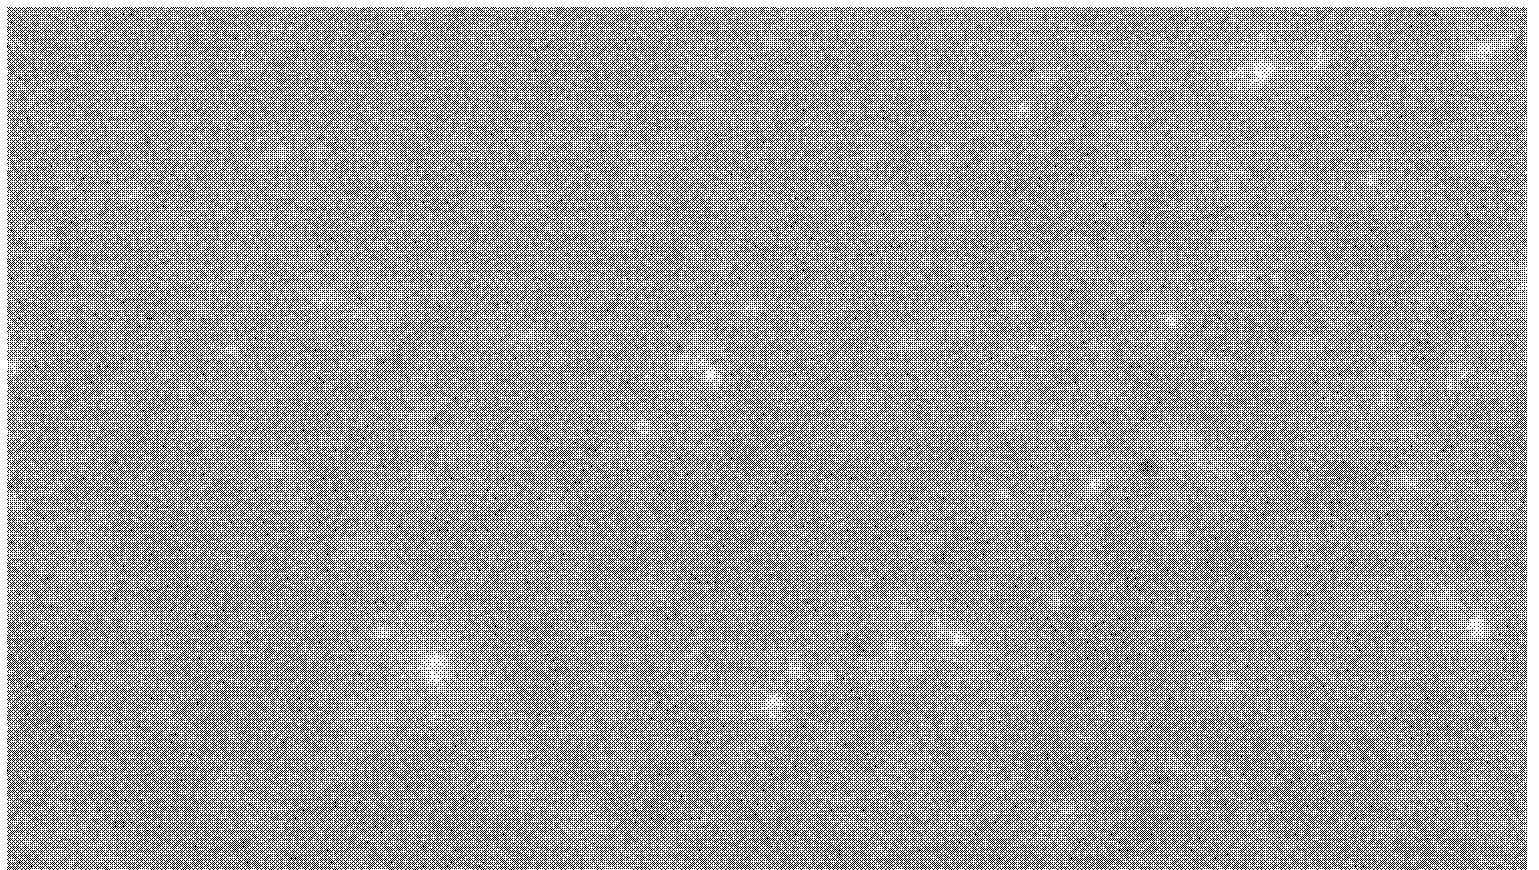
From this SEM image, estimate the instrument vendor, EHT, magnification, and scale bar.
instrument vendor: FEI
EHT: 20 kV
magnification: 5 K X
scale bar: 5000 nm